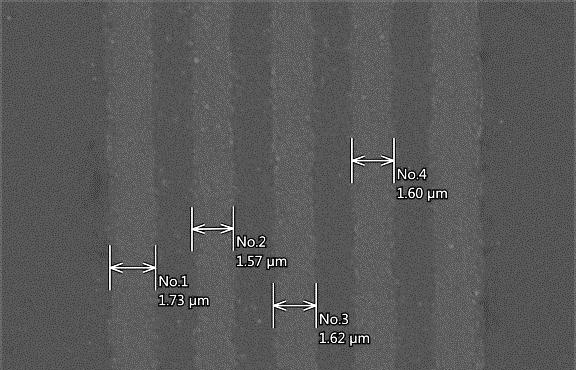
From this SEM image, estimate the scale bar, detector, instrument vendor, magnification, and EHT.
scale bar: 5000 nm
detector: SEI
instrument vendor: JEOL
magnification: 5.5 K X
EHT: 15 kV